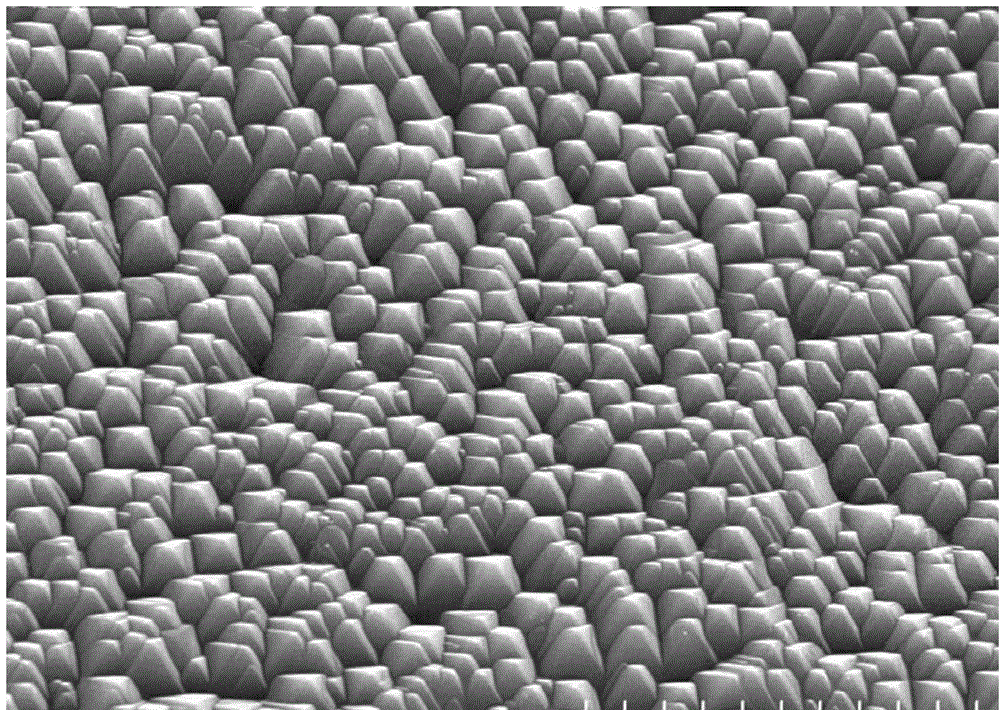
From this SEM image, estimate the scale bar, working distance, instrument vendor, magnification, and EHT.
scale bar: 10000 nm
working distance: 11.4 mm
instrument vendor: Hitachi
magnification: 5 K X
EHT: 15 kV